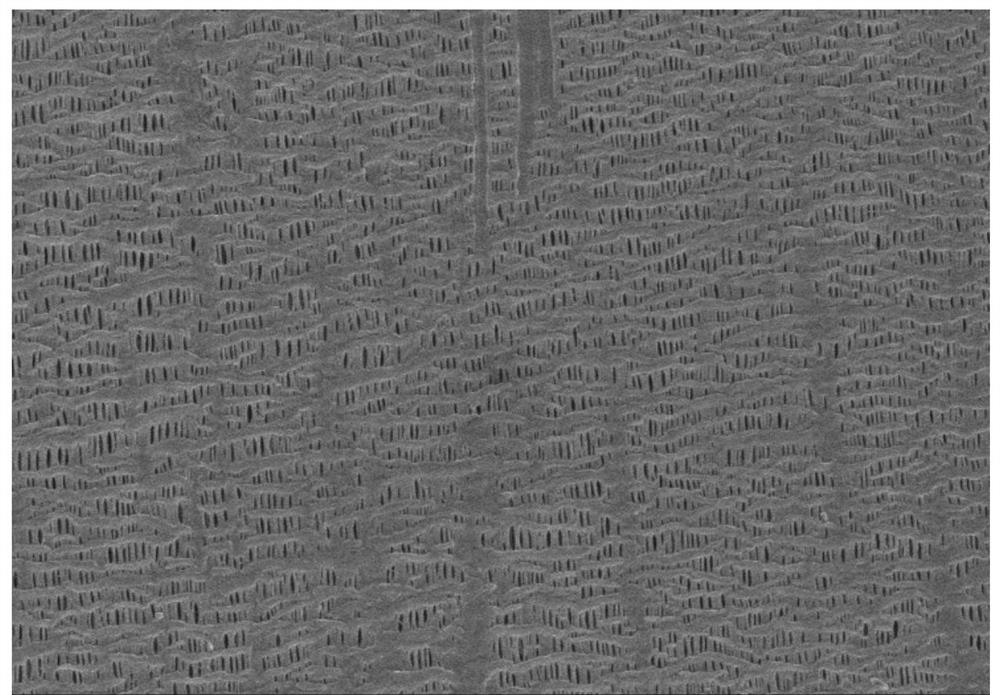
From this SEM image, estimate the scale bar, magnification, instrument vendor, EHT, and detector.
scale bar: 10000 nm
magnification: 5 K X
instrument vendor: Hitachi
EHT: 4 kV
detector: SE(M)